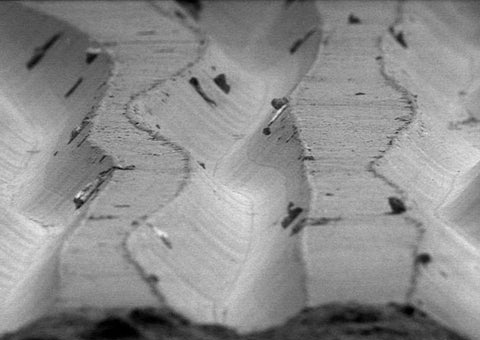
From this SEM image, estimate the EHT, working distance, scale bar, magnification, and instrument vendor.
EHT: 5 kV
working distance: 27 mm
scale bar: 50000 nm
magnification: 0.5 K X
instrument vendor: other